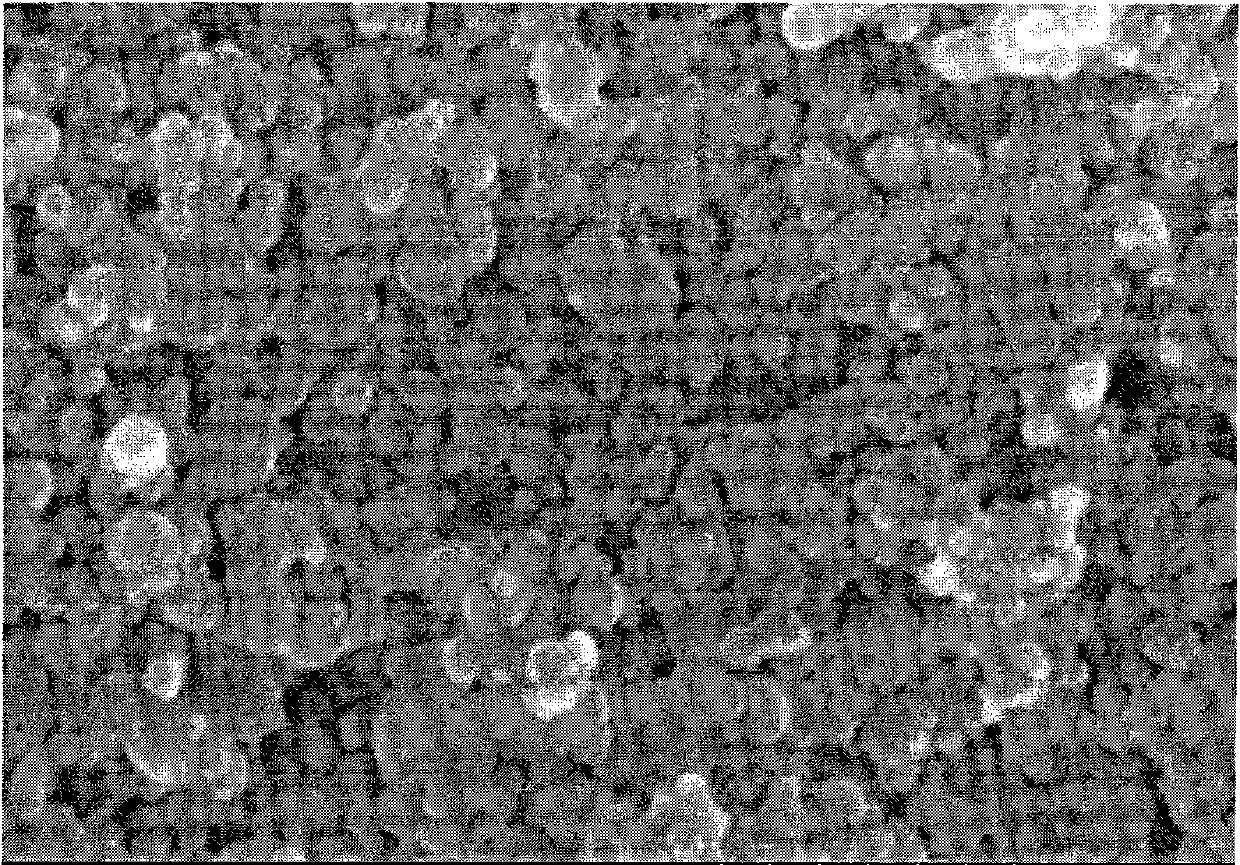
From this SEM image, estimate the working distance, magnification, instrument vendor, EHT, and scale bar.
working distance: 10.3 mm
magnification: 100 K X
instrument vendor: Hitachi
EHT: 20 kV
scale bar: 500 nm